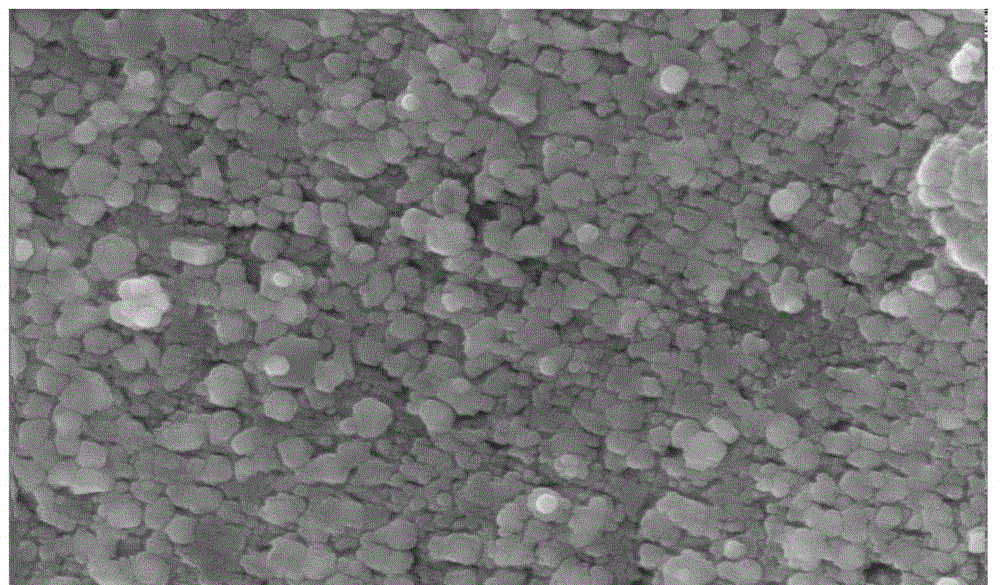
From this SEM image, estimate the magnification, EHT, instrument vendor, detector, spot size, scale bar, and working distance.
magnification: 50 K X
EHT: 20 kV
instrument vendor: FEI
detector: TLD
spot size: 4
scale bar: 1000 nm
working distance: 6.1 mm